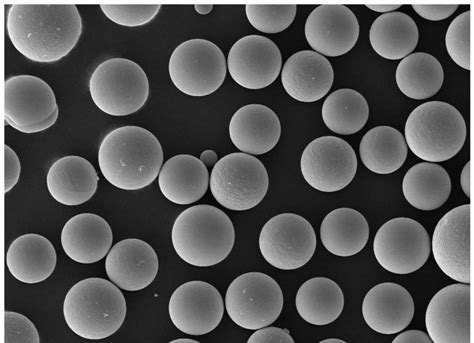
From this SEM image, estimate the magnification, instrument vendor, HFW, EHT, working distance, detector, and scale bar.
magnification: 0.5 K X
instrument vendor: Phenom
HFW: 558 µm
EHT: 15 kV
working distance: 15.21 mm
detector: SE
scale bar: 100000 nm